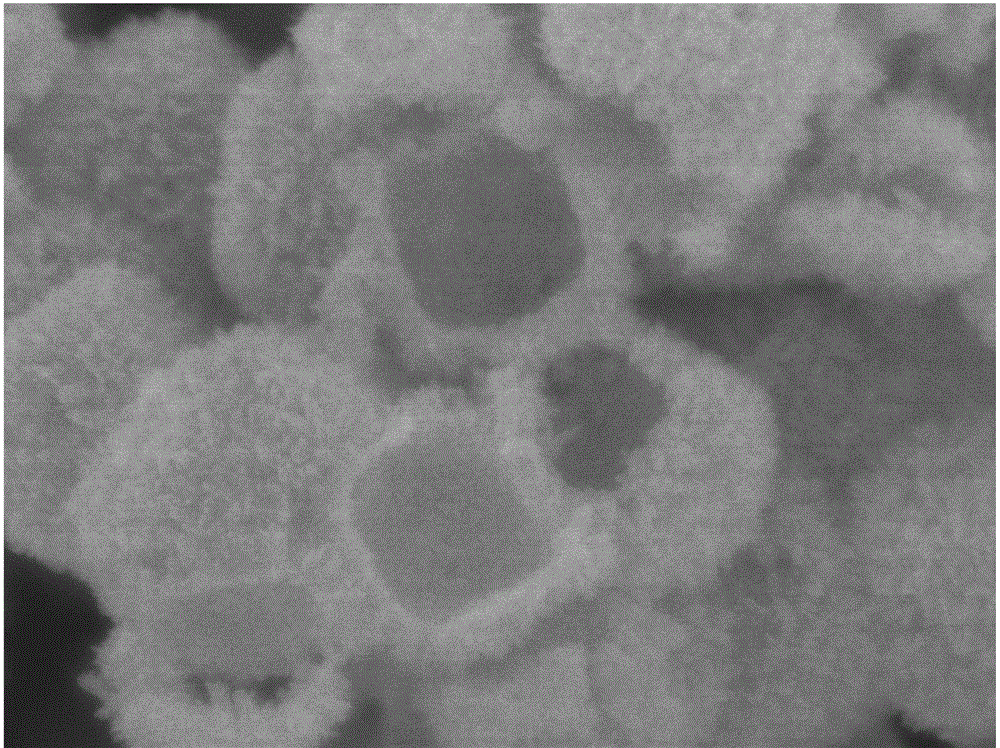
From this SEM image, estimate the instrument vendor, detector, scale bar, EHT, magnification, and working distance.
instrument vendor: JEOL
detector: SEI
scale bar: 100 nm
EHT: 8 kV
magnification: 50 K X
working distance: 6.5 mm